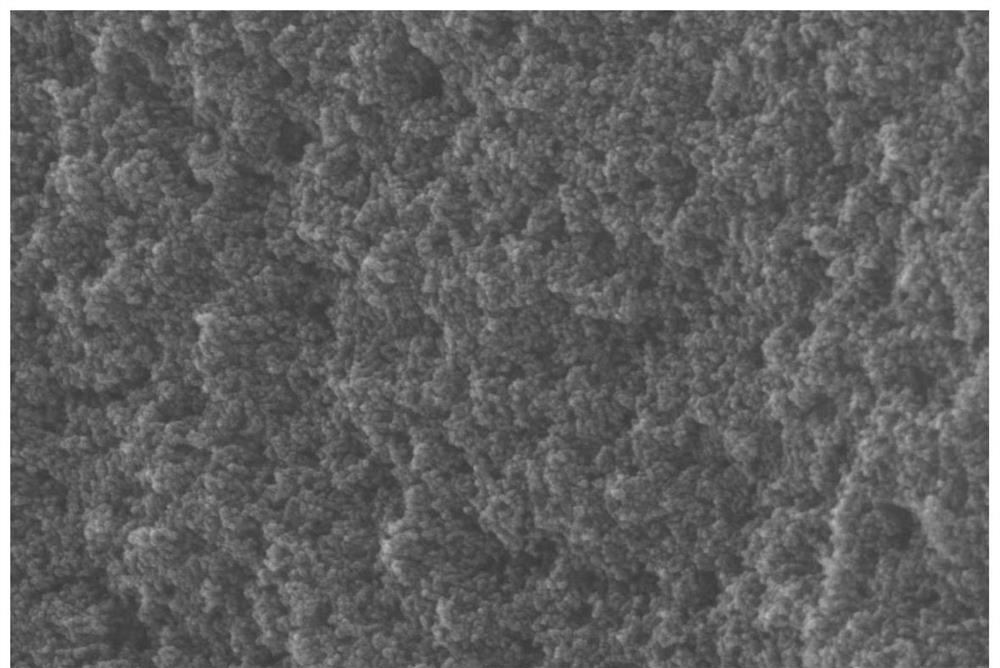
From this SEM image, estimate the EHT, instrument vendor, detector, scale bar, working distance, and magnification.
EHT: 10 kV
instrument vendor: Zeiss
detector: SE2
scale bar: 100 nm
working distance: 14.2 mm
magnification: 40 K X